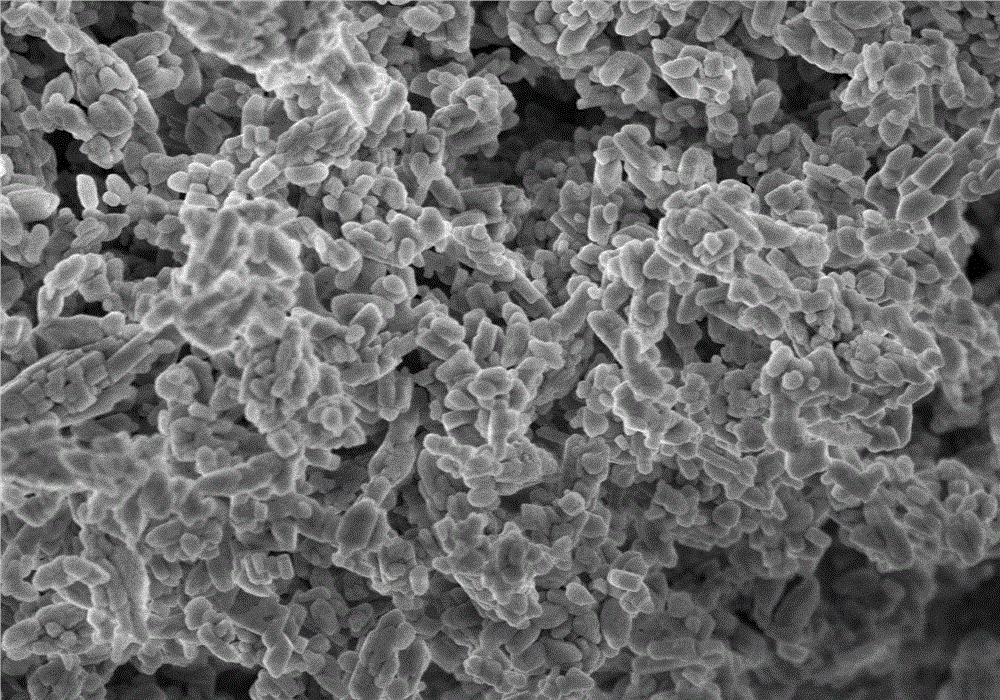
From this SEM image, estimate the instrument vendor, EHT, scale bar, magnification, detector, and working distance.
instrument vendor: Hitachi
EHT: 3 kV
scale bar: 2000 nm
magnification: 20 K X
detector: SE(M,LA0)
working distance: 3.9 mm